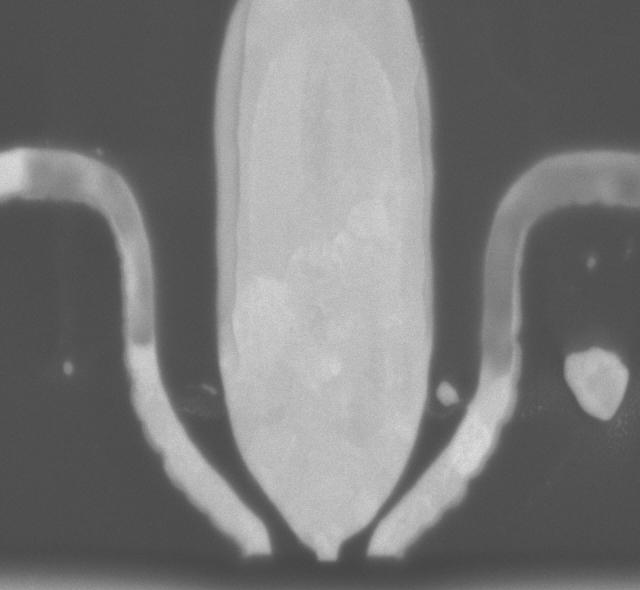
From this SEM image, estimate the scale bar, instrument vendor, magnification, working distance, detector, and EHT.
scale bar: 500 nm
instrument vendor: Hitachi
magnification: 100 K X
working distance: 1 mm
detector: YAG-BSE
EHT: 5 kV